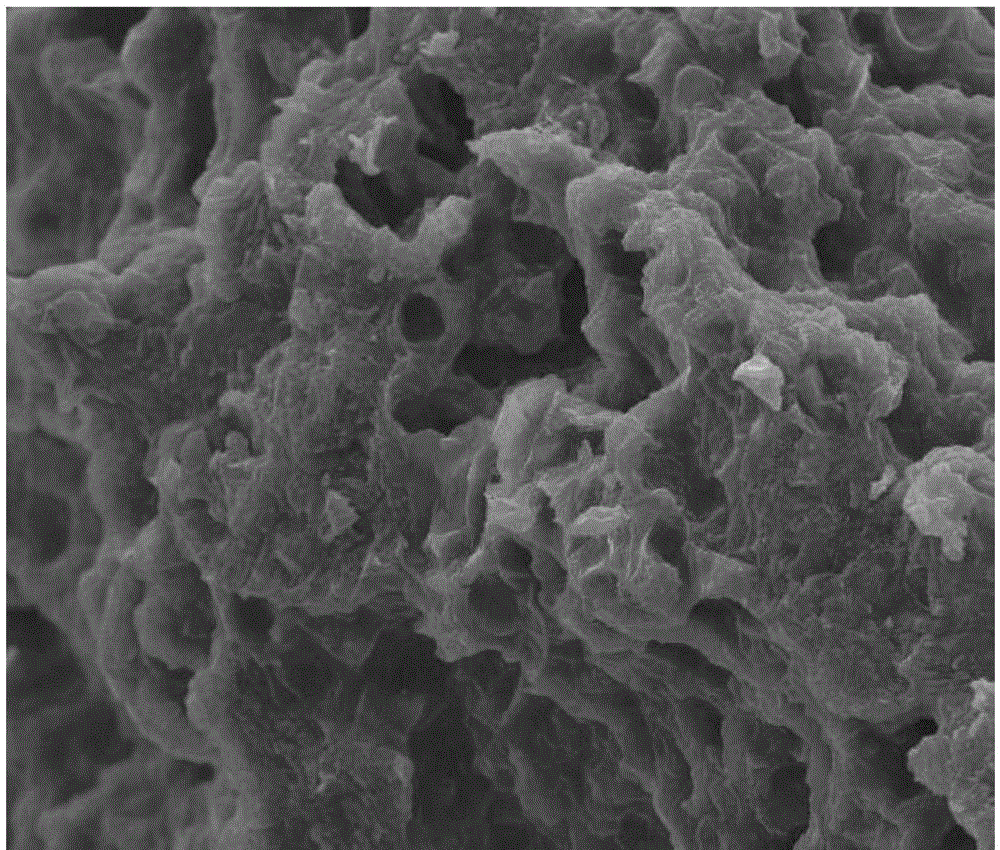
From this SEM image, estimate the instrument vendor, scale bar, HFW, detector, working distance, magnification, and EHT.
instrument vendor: FEI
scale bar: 50000 nm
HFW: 270 µm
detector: ETD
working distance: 9 mm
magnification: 1 K X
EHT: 20 kV